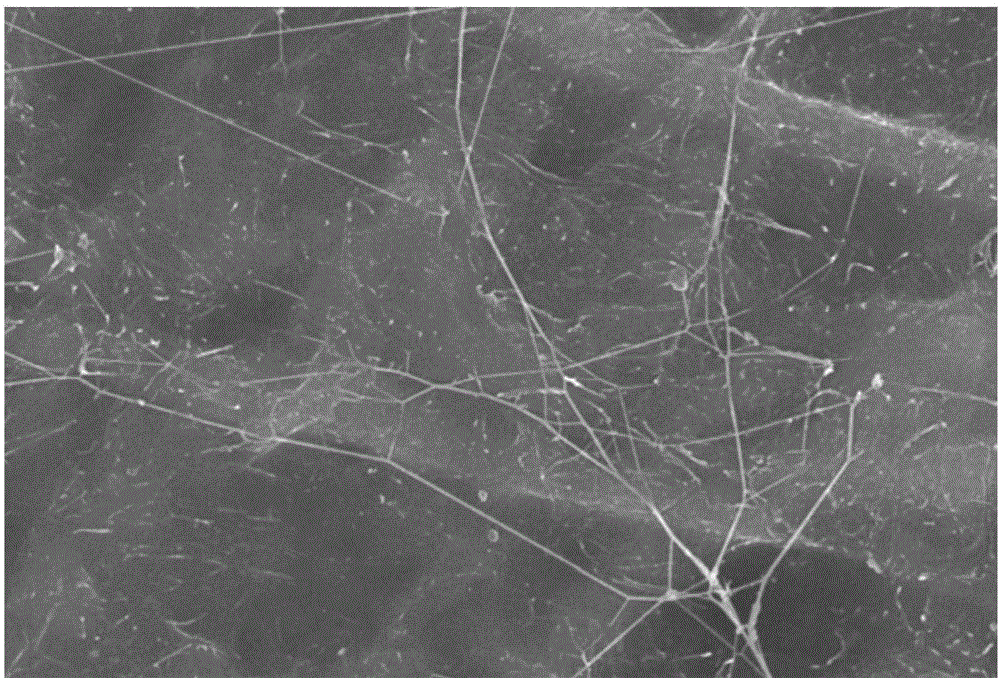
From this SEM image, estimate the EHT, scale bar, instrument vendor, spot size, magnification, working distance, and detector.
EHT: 20 kV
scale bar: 5000 nm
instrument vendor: JEOL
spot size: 40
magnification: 5 K X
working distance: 13 mm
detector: SEI